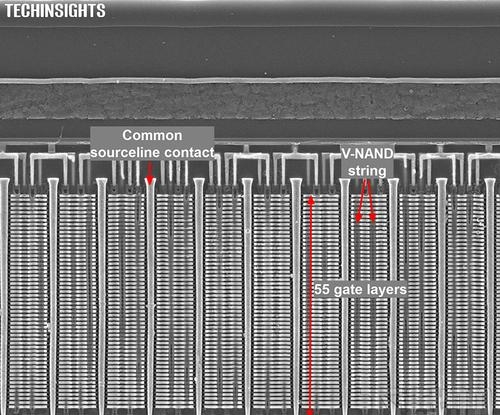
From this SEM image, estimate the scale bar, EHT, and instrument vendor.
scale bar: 1000 nm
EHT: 5 kV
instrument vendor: Zeiss